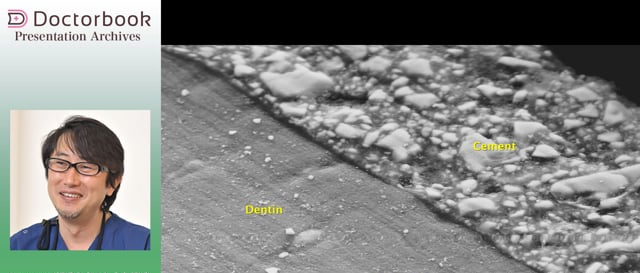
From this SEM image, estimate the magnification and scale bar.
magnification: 1.5 K X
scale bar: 20000 nm